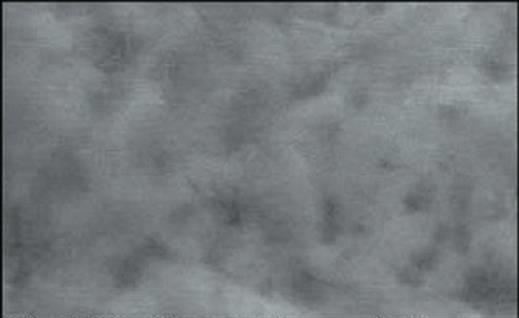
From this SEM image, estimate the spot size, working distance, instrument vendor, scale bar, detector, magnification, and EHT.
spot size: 3.9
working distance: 8.4 mm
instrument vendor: FEI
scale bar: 5000 nm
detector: GSE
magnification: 4 K X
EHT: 20 kV